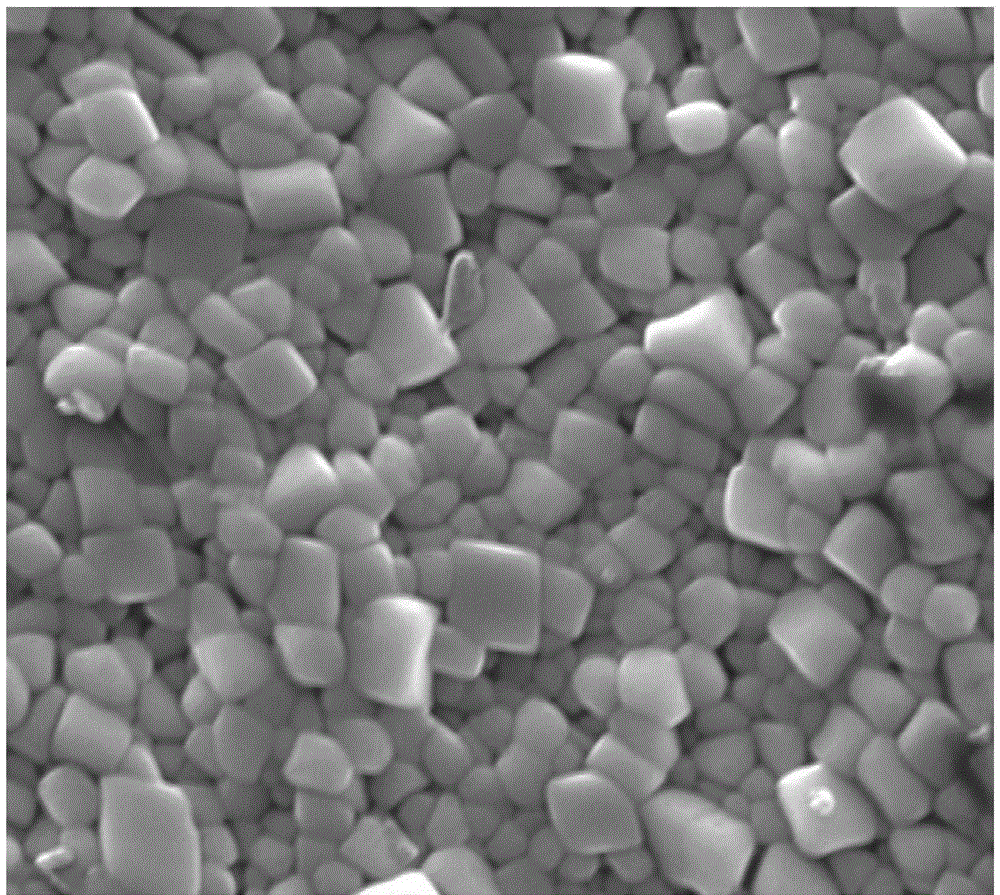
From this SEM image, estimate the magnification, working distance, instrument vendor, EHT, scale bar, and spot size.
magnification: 10 K X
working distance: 22.8 mm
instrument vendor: FEI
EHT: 20 kV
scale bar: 4000 nm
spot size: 4